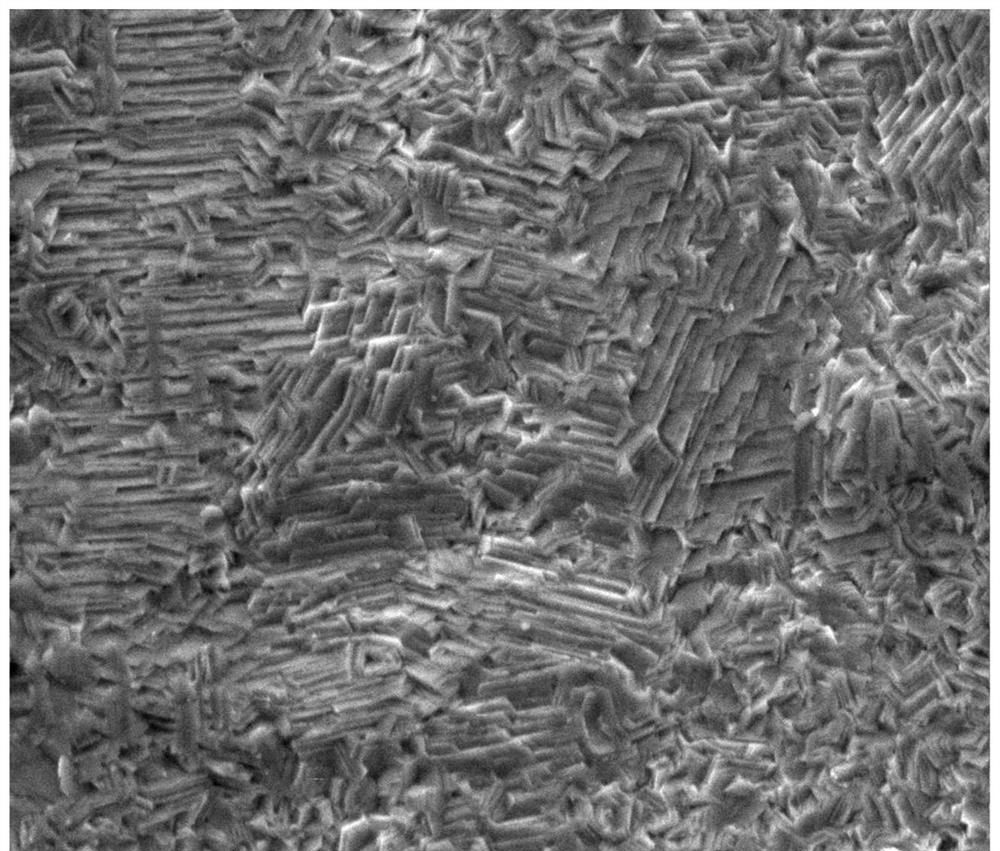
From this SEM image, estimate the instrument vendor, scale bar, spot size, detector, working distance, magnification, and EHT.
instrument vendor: FEI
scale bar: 20000 nm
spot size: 4.5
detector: ETD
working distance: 12.1 mm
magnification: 2 K X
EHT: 20 kV